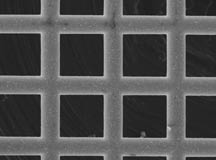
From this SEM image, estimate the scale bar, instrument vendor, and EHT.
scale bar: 100000 nm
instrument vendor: JEOL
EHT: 15 kV